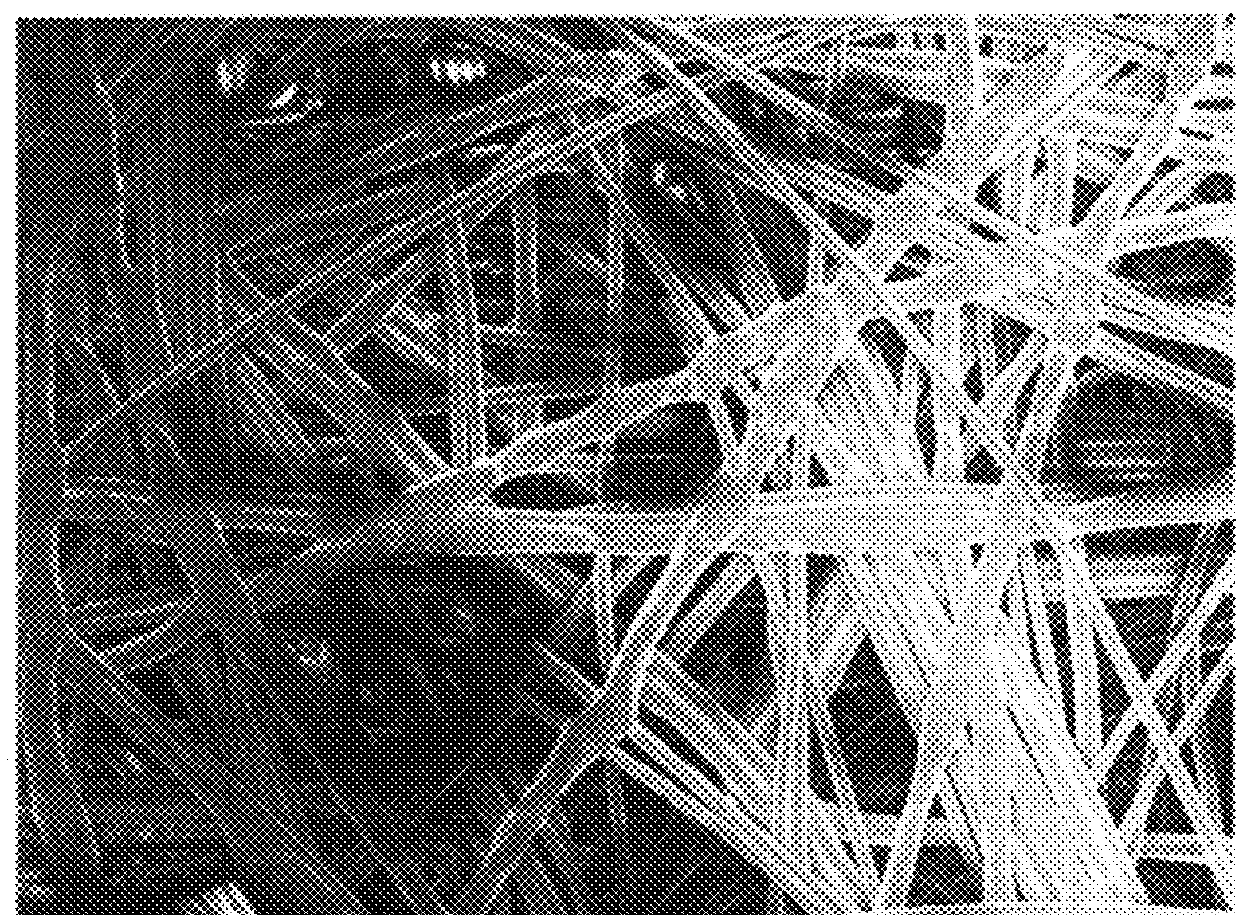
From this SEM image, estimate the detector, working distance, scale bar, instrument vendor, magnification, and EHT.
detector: SEI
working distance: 10 mm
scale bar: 100000 nm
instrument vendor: JEOL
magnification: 0.2 K X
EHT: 5 kV